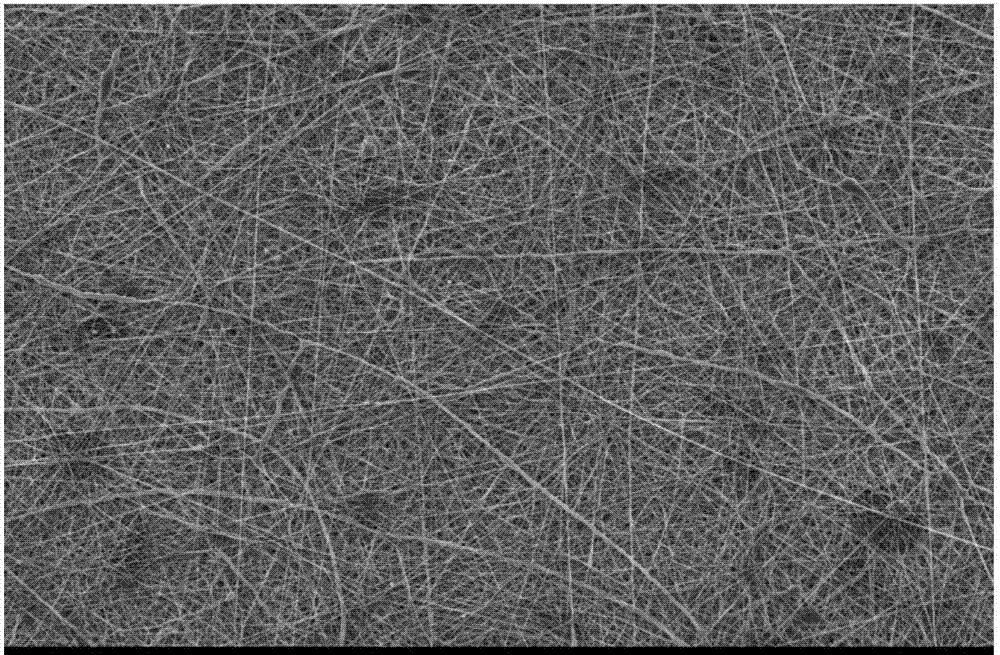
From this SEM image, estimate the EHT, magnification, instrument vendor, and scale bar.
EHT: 10 kV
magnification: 0.5 K X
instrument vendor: JEOL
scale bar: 50000 nm